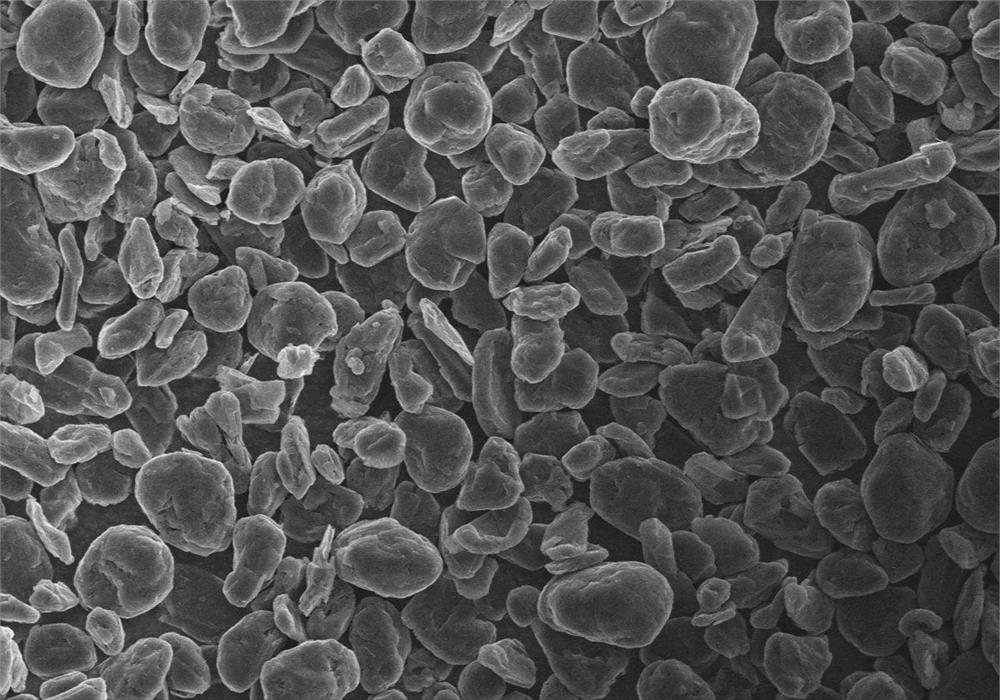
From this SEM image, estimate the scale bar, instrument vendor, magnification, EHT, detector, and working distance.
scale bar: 100000 nm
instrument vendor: Hitachi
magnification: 0.5 K X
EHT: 15 kV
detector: SE(M)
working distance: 8.3 mm